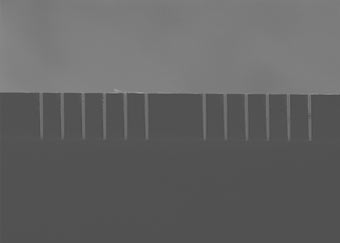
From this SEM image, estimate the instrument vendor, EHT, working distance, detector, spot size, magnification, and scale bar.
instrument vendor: FEI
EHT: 5 kV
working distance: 4.7 mm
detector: SE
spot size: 3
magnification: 0.383 K X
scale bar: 50000 nm